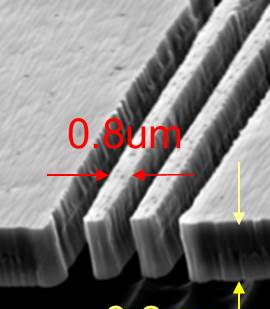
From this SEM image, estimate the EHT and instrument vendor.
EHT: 15 kV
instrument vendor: JEOL